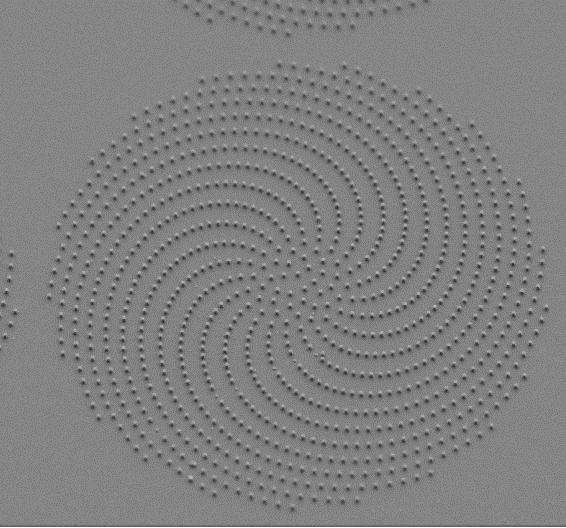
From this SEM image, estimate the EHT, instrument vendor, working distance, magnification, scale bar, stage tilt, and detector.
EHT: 2 kV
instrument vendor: Zeiss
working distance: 5.6 mm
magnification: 2 K X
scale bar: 2000 nm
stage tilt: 45°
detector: SE2